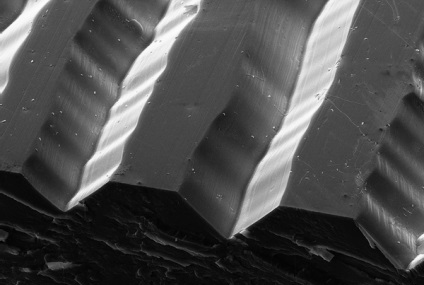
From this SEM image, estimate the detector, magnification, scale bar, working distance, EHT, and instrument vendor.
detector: SE2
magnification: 0.34 K X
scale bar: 10000 nm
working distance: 11.1 mm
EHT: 20 kV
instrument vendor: Zeiss